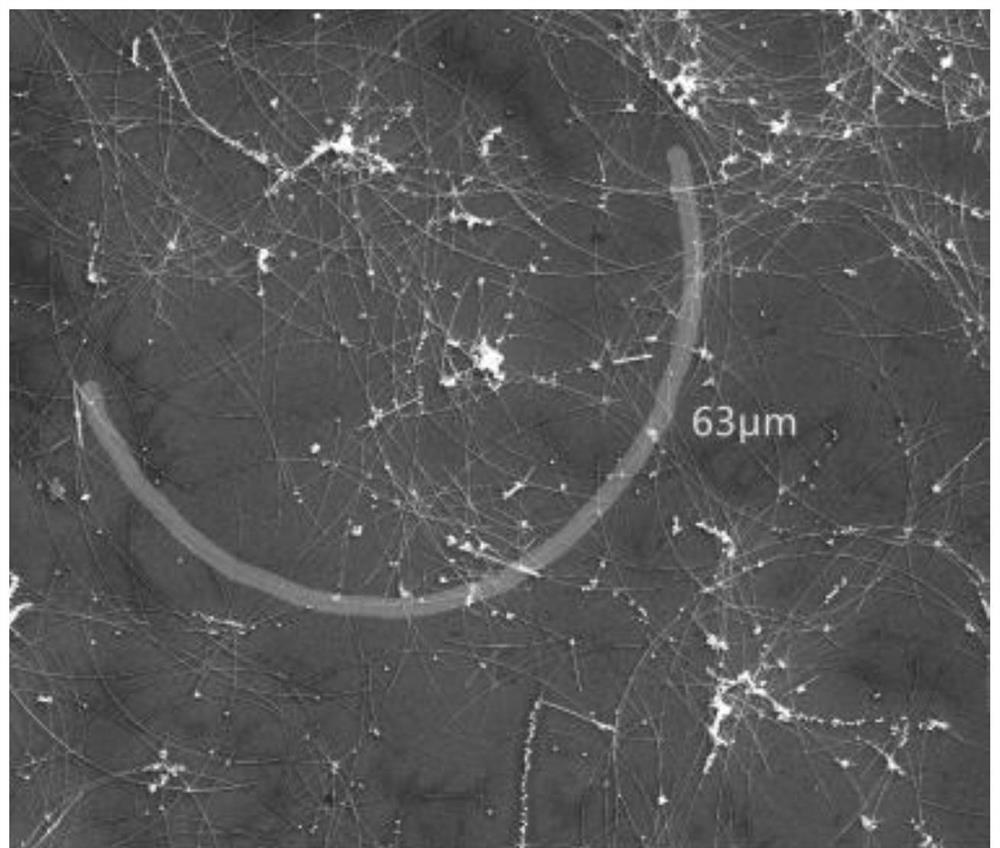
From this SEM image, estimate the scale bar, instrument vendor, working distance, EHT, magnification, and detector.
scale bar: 10000 nm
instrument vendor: FEI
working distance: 6.4 mm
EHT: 20 kV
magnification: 5 K X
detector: SE